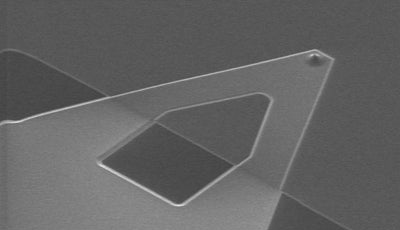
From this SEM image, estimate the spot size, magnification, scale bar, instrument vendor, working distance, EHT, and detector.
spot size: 3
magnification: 0.5 K X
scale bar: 50000 nm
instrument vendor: FEI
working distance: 3.4 mm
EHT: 10 kV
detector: SE